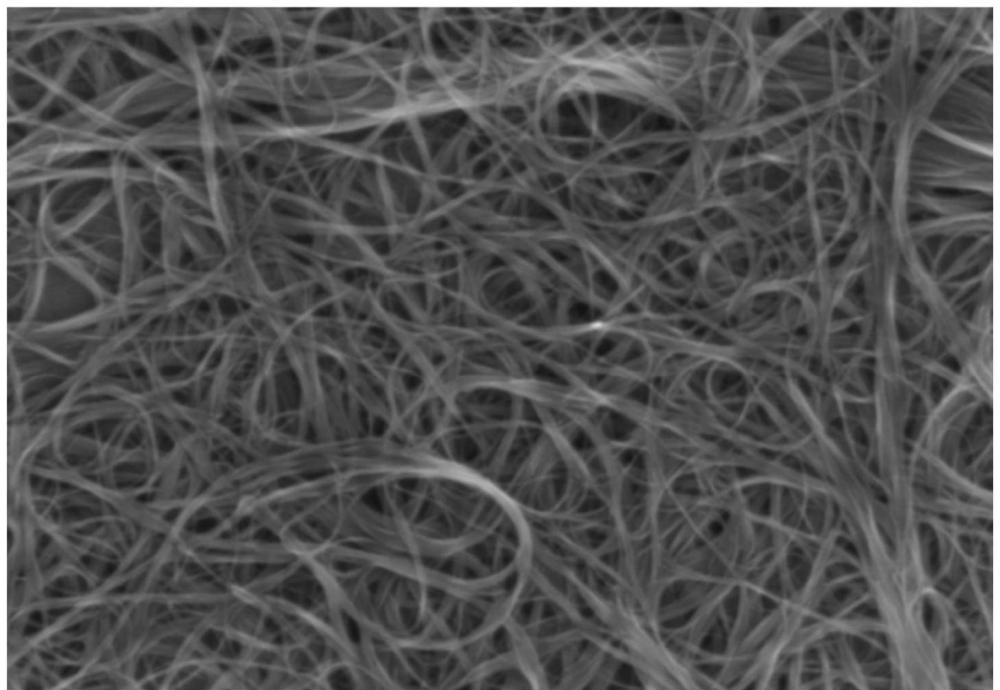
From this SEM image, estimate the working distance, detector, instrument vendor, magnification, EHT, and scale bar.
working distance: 12.3 mm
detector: SE(U)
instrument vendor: Hitachi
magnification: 50 K X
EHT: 3 kV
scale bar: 1000 nm